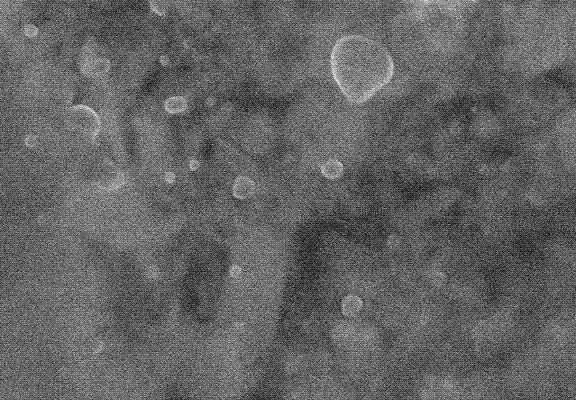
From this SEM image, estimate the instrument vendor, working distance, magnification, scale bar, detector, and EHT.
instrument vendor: Hitachi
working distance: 10.4 mm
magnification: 50 K X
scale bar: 1000 nm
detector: SE(M)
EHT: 5 kV